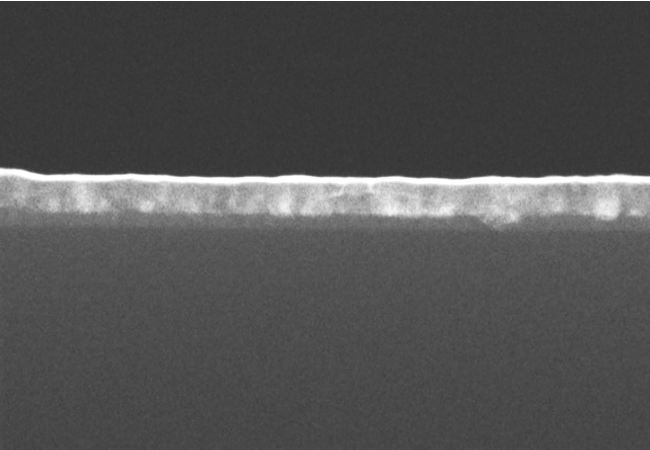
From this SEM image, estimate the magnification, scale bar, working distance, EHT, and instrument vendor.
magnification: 150 K X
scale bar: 300 nm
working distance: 8.2 mm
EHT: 10 kV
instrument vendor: Hitachi